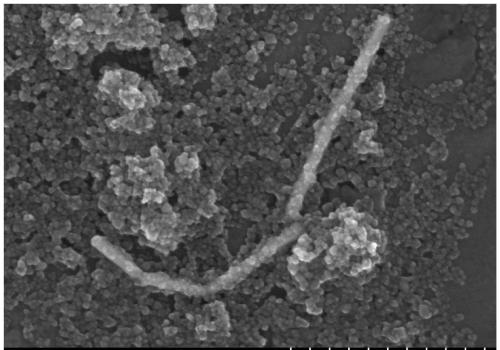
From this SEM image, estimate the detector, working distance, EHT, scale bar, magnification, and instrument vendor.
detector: SE(U)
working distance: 2.8 mm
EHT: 5 kV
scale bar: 500 nm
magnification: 100 K X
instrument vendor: Hitachi